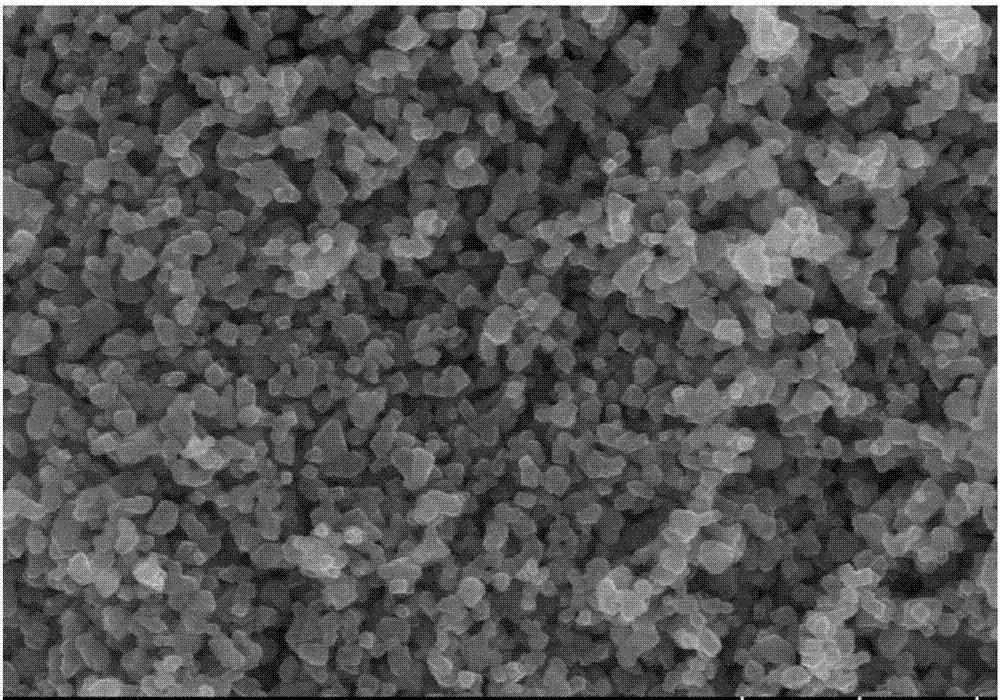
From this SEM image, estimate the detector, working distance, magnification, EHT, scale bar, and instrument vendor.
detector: SE(UL)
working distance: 9.8 mm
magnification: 30 K X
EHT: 15 kV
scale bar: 1000 nm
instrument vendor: Hitachi